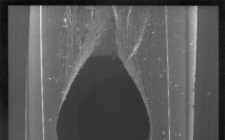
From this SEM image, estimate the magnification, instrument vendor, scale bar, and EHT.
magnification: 0.015 K X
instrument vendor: JEOL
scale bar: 1000000 nm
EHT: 25 kV